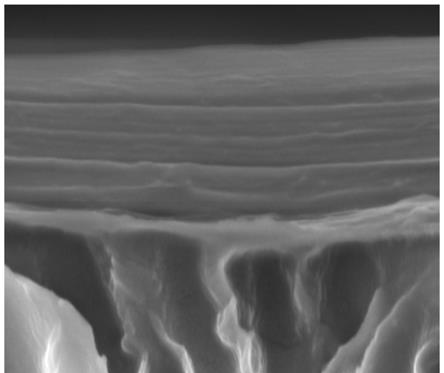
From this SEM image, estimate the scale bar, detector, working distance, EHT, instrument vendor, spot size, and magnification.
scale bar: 1000 nm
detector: ETD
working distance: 9.7 mm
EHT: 8 kV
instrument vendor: FEI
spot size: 3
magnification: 80 K X